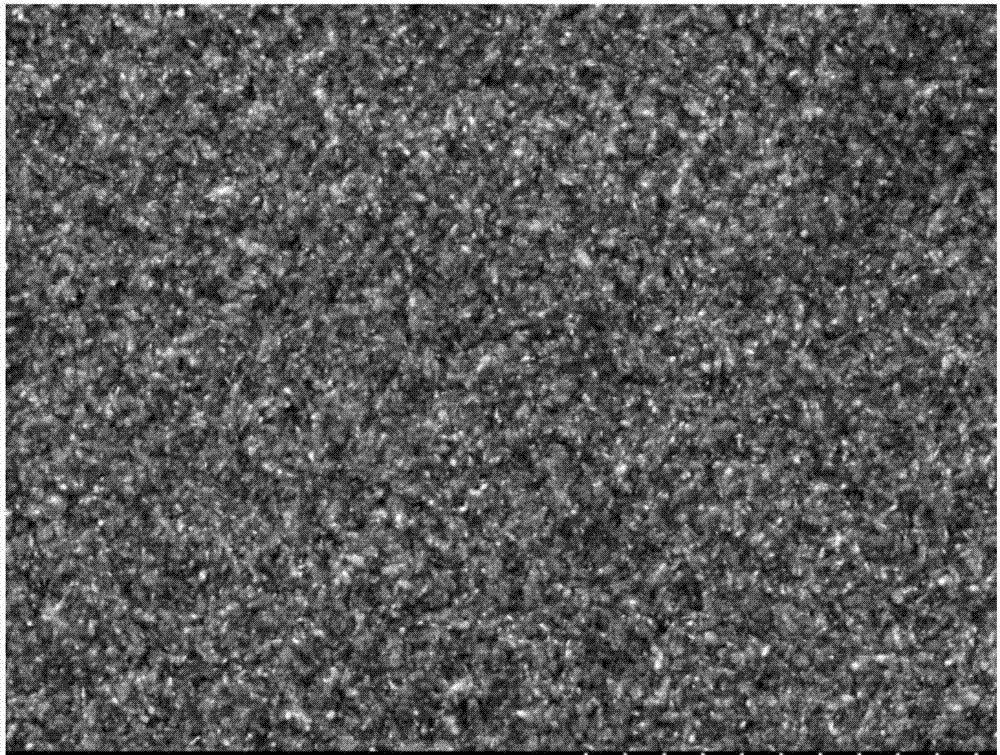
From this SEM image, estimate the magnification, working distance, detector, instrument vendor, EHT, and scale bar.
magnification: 5 K X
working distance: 15.9 mm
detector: SE(M)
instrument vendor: Hitachi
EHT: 20 kV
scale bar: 10000 nm